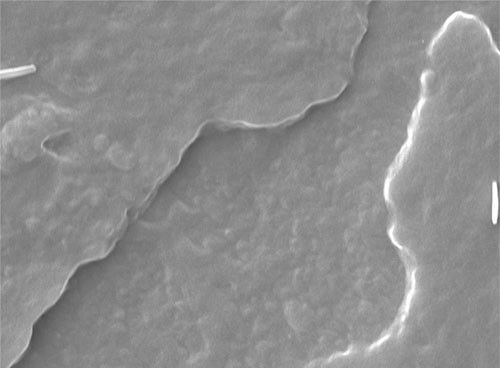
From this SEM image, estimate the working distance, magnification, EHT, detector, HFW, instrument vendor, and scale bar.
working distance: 11.6 mm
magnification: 7.5 K X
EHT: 10 kV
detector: BD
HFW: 17.1 µm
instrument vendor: Hitachi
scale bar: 1000 nm